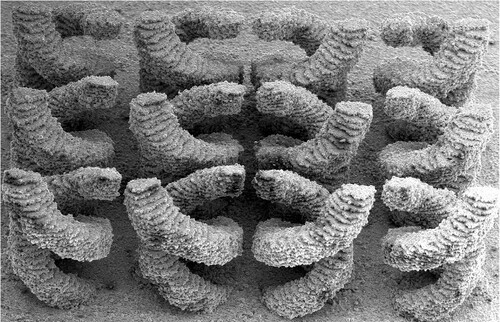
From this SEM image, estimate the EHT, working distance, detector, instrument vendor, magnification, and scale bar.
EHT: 5 kV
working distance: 23.6 mm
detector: ETD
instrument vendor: FEI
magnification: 0.065 K X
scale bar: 1000000 nm